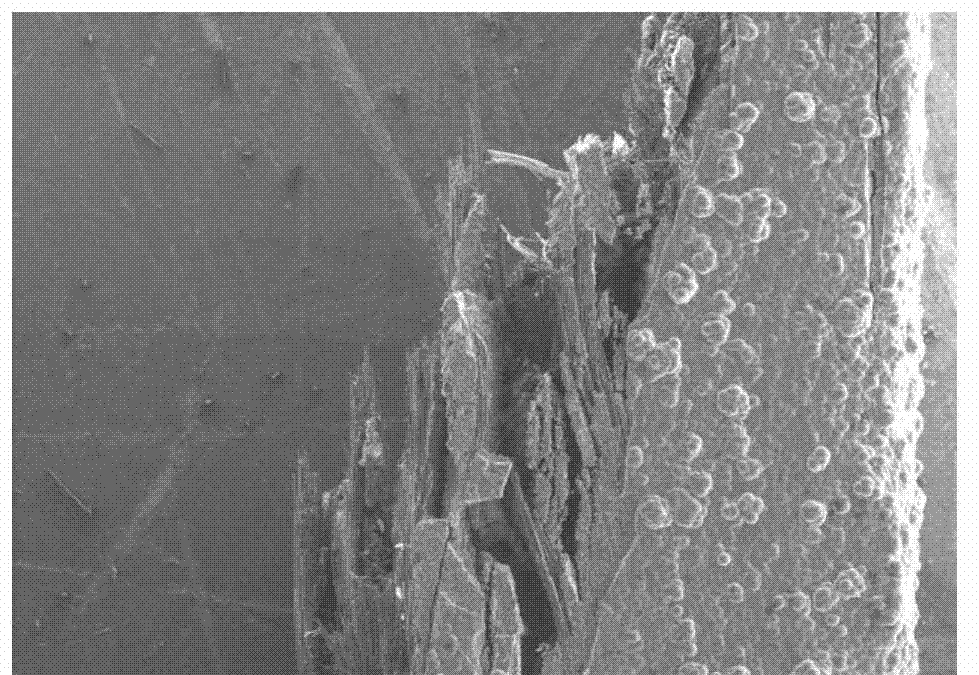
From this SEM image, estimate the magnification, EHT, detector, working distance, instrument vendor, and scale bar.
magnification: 0.035 K X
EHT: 15 kV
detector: SE(M)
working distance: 15.5 mm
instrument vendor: Hitachi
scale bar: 1000000 nm